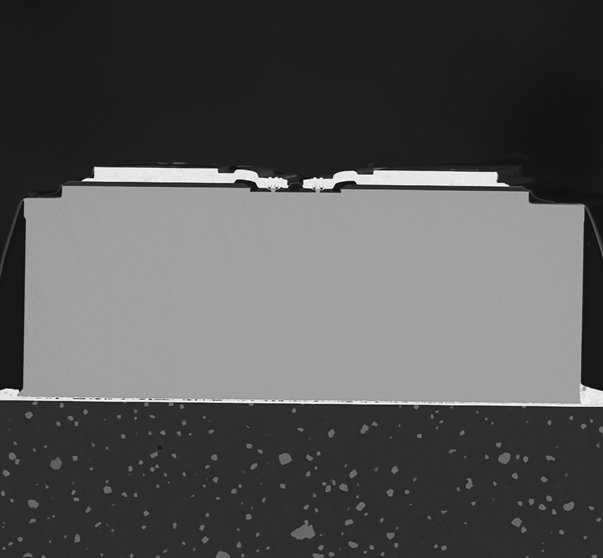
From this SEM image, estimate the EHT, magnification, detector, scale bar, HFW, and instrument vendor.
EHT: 15 kV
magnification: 1 K X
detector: BSD Full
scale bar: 80000 nm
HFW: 269 µm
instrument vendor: other